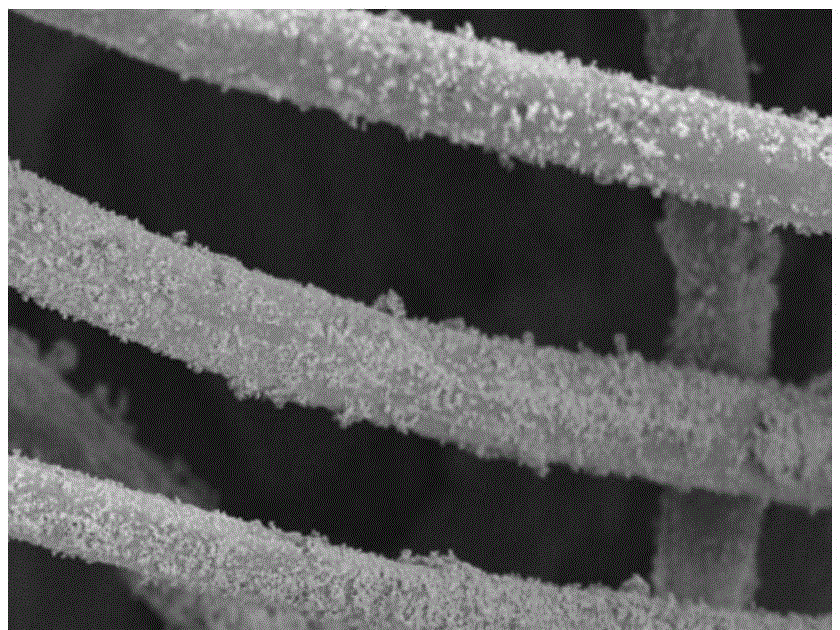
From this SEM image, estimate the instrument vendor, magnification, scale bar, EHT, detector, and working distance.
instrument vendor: Hitachi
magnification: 1 K X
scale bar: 50000 nm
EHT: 5 kV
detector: SE(UL)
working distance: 12.2 mm